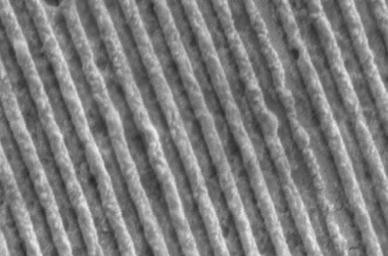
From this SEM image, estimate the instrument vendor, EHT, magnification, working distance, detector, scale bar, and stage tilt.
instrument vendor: Zeiss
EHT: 5 kV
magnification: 24.29 K X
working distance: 16.1 mm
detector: SE2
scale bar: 100 nm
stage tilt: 8.8°